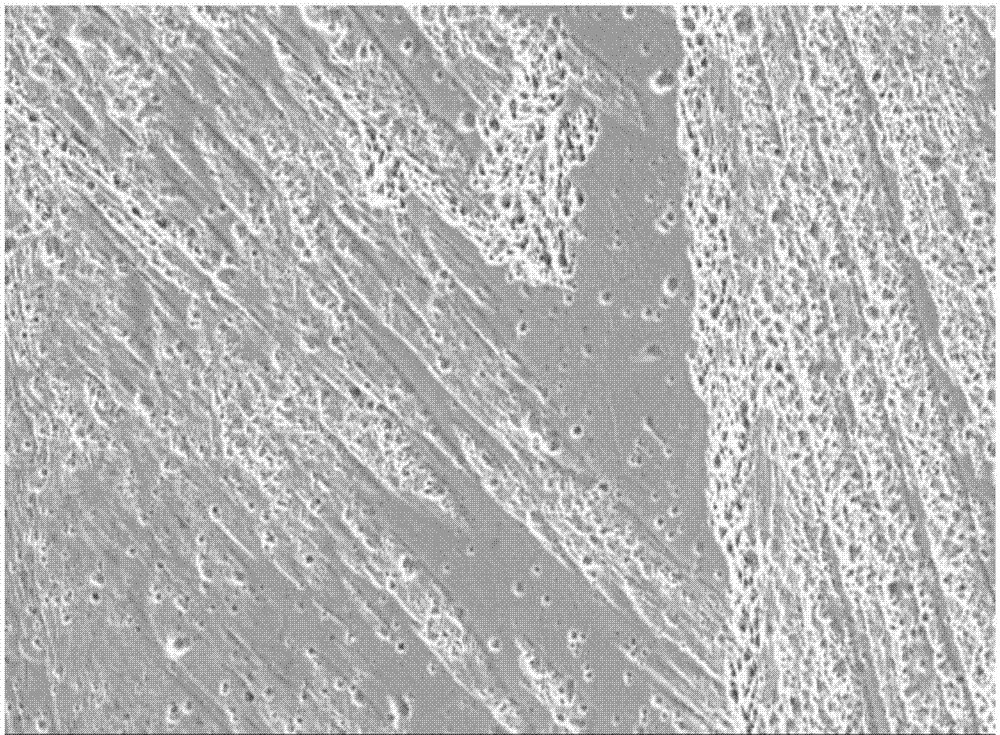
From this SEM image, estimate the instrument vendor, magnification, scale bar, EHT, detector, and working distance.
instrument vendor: Hitachi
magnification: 0.5 K X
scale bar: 100000 nm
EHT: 20 kV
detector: SE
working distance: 11.3 mm